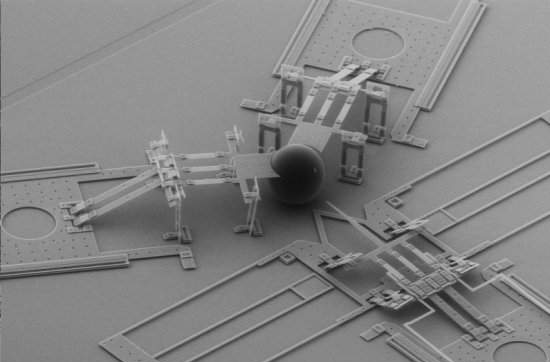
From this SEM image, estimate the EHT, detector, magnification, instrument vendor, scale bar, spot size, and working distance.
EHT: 15 kV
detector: GSE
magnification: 0.137 K X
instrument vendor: FEI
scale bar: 200000 nm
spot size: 3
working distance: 12.7 mm